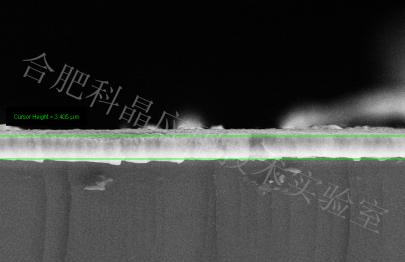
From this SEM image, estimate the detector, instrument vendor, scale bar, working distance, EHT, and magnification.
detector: SE2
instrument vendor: Zeiss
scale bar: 2000 nm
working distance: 8.5 mm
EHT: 20 kV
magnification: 2 K X